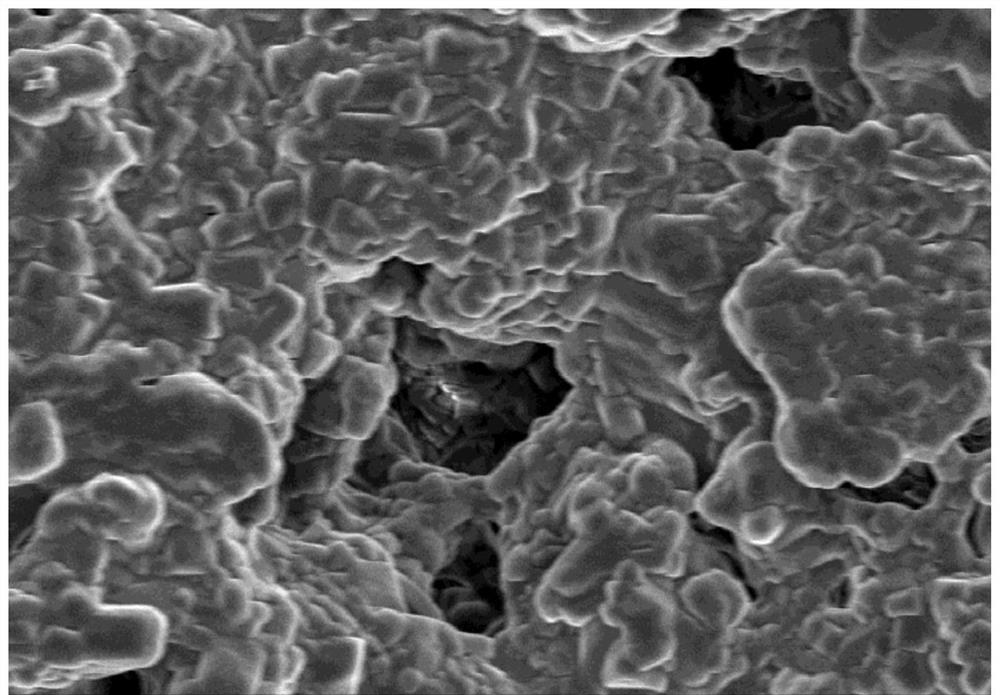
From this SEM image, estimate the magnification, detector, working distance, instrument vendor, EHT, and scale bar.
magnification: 5 K X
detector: SE(M)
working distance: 9.5 mm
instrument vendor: Hitachi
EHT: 5 kV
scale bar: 10000 nm